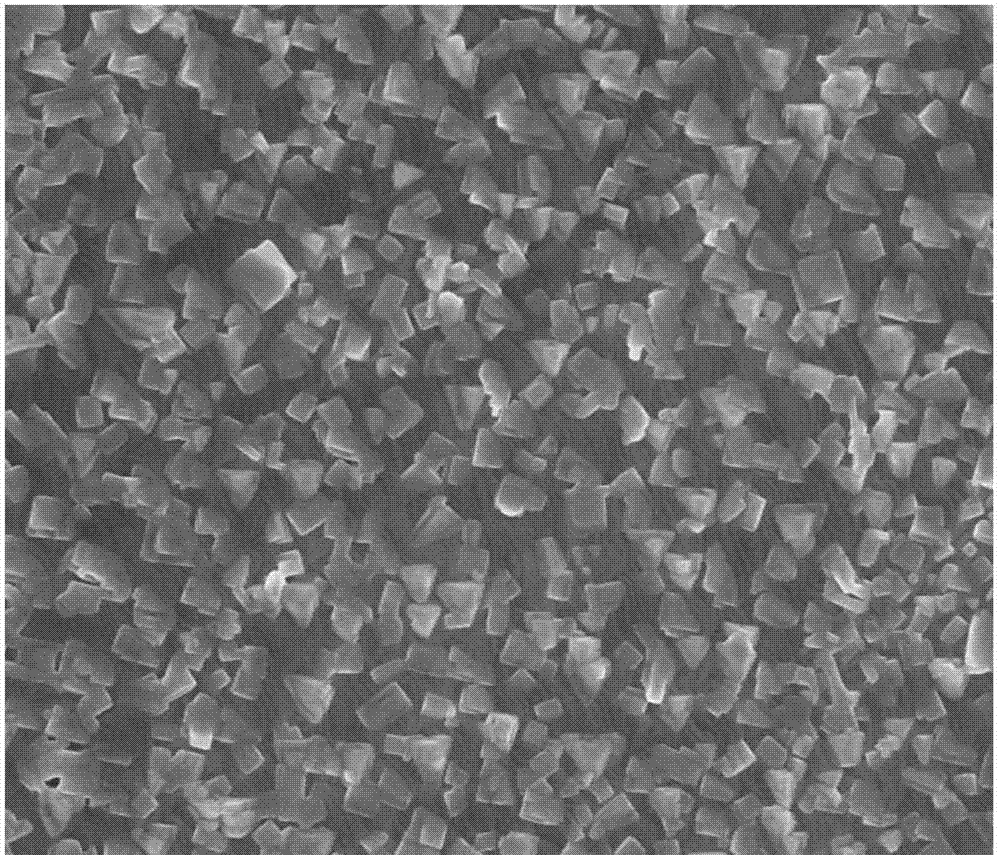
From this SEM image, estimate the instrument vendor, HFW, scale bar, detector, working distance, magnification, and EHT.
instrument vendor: FEI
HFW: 14.9 µm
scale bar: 5000 nm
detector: TLD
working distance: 5.1 mm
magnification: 20 K X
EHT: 10 kV